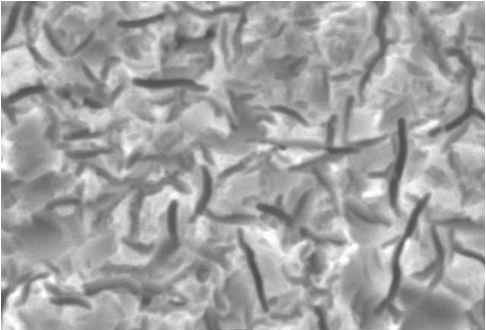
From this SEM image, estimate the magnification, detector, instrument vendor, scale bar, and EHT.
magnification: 3 K X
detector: SEI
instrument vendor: JEOL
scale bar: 5000 nm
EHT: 20 kV